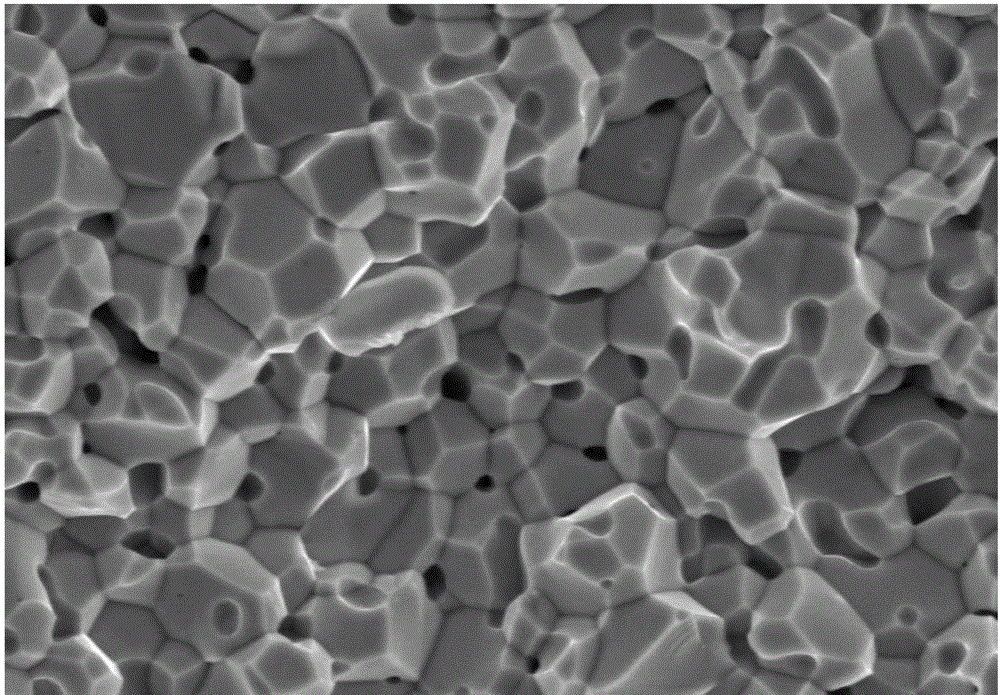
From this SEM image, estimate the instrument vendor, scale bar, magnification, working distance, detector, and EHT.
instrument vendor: JEOL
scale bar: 10000 nm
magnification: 2 K X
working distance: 8 mm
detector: SEI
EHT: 15 kV